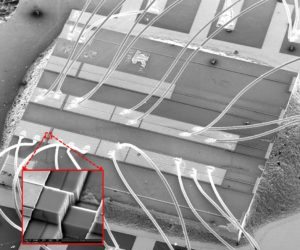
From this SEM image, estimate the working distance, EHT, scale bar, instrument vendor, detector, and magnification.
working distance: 10.8 mm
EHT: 15 kV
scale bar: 1000000 nm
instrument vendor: FEI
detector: ETD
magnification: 0.085 K X